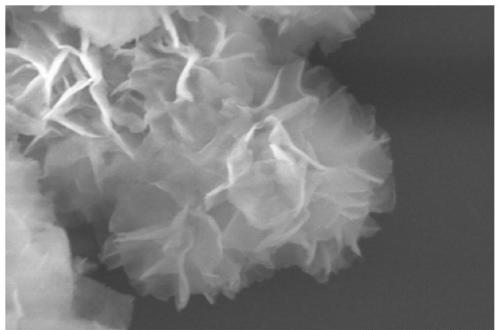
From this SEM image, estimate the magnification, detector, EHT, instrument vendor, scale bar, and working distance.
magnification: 30 K X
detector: SE(M)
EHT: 10 kV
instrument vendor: Hitachi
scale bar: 1000 nm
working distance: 7.9 mm